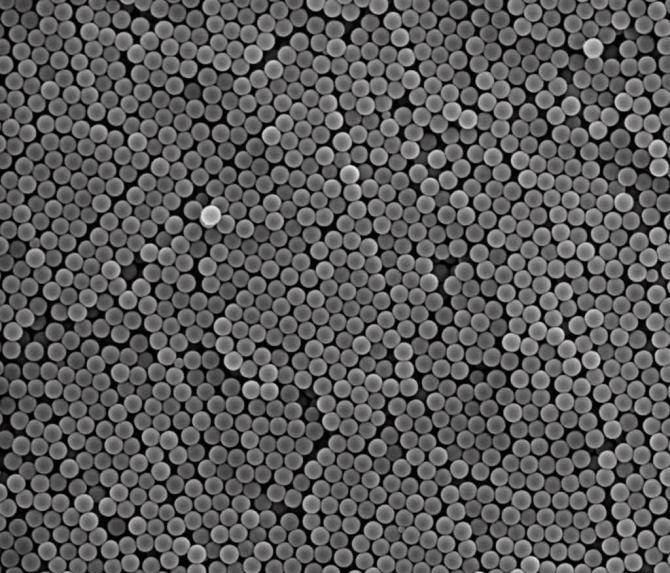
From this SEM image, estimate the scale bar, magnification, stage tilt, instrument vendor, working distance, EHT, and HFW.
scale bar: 5000 nm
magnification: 20 K X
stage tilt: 0°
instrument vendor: FEI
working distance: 10 mm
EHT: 25 kV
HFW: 13.5 µm